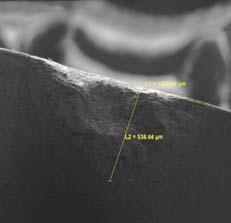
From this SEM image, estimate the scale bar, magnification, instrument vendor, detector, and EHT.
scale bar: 500000 nm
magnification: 0.172 K X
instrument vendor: Tescan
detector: SE Detector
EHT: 5 kV